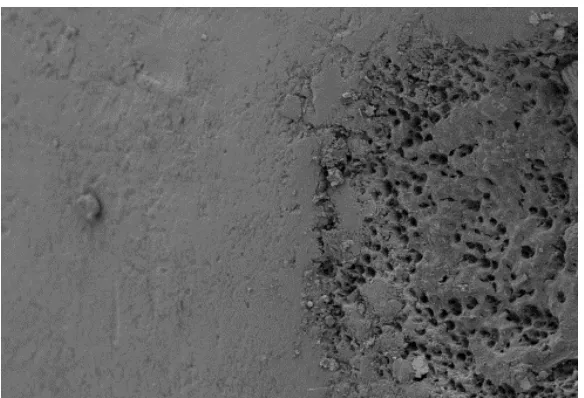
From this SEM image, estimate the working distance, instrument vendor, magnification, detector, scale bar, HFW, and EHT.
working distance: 9.4 mm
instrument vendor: Zeiss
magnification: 1 K X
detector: SE2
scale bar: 10000 nm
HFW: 301.6 µm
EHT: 5 kV